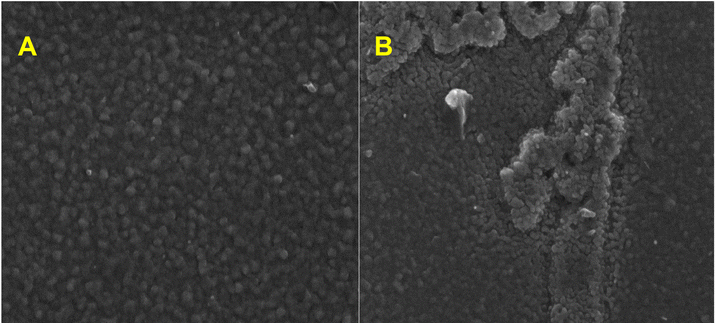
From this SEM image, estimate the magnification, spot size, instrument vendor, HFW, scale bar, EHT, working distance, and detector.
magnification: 50 K X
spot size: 4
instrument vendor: FEI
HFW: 5.97 µm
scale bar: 2000 nm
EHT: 5 kV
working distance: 5.3 mm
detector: TLD